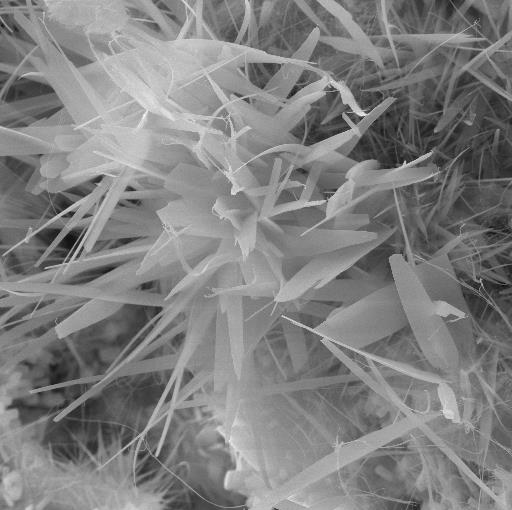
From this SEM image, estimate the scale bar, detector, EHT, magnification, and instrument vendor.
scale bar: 5000 nm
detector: SE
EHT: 20 kV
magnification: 5 K X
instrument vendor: Tescan